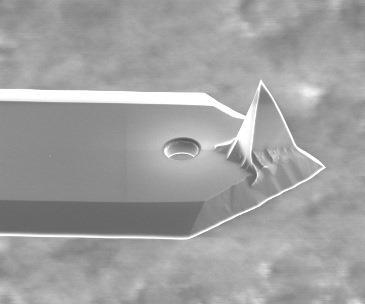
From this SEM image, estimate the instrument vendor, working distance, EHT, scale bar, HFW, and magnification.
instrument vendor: FEI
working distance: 3.8 mm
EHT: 5 kV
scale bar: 20000 nm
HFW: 64 µm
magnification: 4 K X